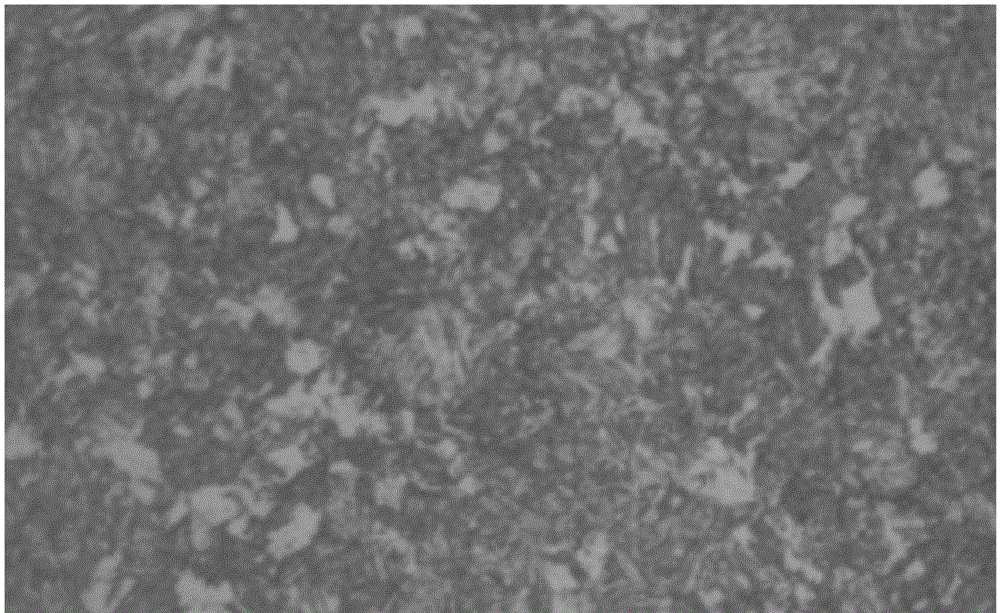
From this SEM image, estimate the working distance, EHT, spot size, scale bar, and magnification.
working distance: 7.9 mm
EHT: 2 kV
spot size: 3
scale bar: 10000 nm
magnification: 8 K X